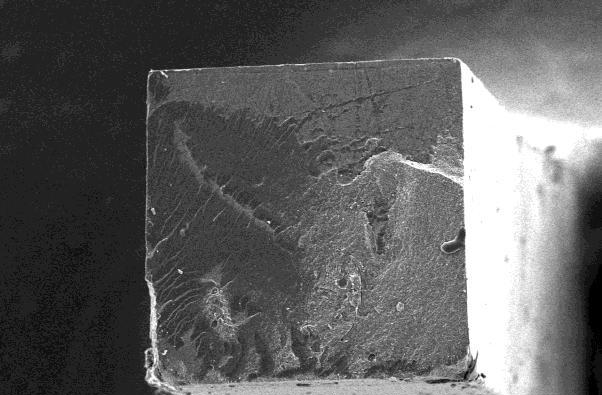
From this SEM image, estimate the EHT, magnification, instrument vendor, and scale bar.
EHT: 15 kV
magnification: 0.06 K X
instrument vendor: JEOL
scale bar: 200000 nm